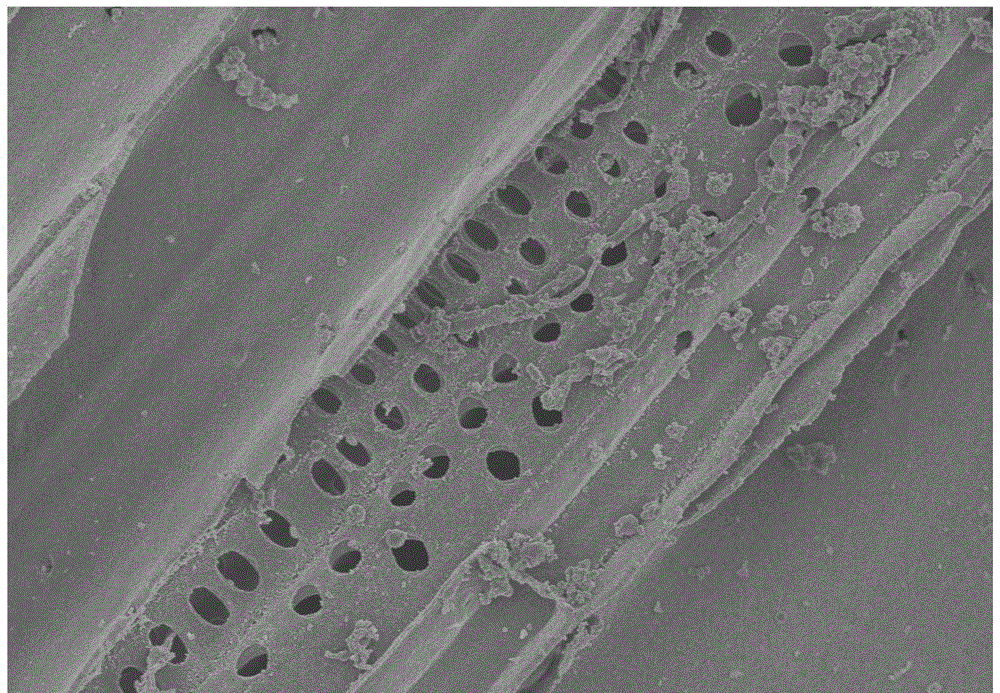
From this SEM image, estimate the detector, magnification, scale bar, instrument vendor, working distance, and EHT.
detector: SE(M)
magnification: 2 K X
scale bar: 20000 nm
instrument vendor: Hitachi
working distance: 8.3 mm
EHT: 3 kV